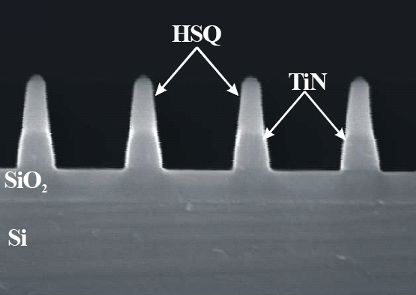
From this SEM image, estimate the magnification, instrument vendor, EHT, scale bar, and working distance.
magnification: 100 K X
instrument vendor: JEOL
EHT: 10 kV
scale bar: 200 nm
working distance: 4 mm